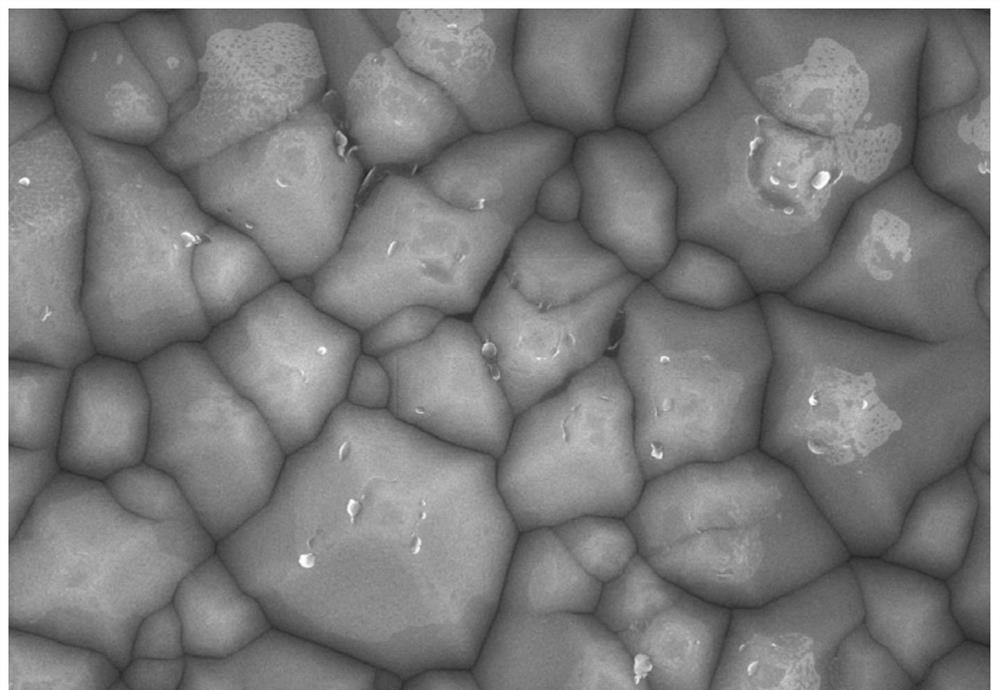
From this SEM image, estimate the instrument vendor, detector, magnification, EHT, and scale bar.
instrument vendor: Hitachi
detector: SE(U)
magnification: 10 K X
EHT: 10 kV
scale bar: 5000 nm